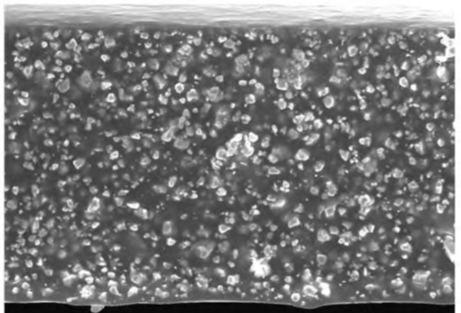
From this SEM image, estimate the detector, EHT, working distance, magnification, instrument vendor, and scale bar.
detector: SE(U)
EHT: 20 kV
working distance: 9.1 mm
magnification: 0.9 K X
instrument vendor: Hitachi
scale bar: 50000 nm